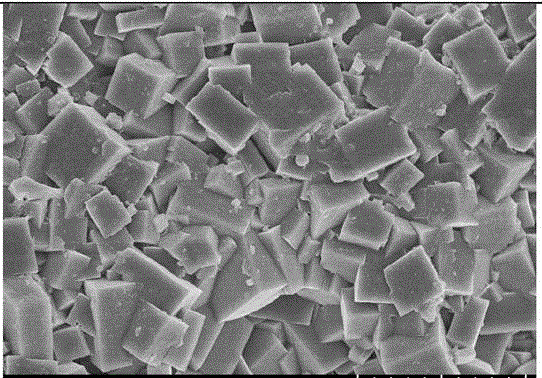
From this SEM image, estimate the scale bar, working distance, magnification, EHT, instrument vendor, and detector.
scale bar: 5000 nm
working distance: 10.6 mm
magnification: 8 K X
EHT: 5 kV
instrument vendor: Hitachi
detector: SE(UL)